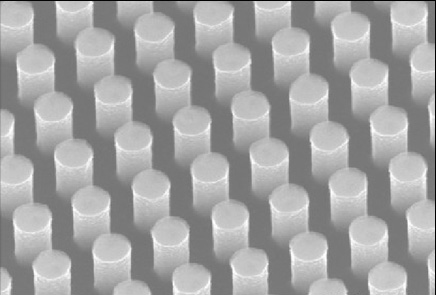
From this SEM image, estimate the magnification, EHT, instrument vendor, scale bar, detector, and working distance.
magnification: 60 K X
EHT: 10 kV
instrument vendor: Hitachi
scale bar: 500 nm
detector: SE(U)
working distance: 10.9 mm